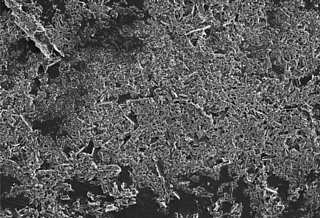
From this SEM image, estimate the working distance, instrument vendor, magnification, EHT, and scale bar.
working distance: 4.5 mm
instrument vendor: Hitachi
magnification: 0.5 K X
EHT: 5 kV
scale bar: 20000 nm